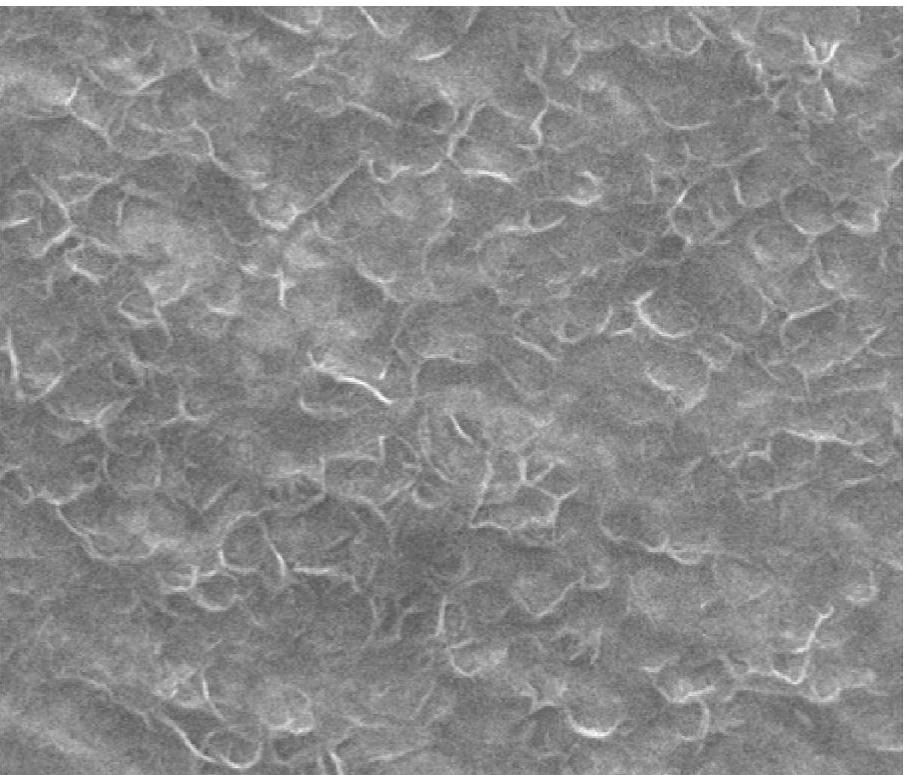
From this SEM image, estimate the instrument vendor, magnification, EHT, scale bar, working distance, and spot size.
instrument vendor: FEI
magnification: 100 K X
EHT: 20 kV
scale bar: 1000 nm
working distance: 11 mm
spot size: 2.5